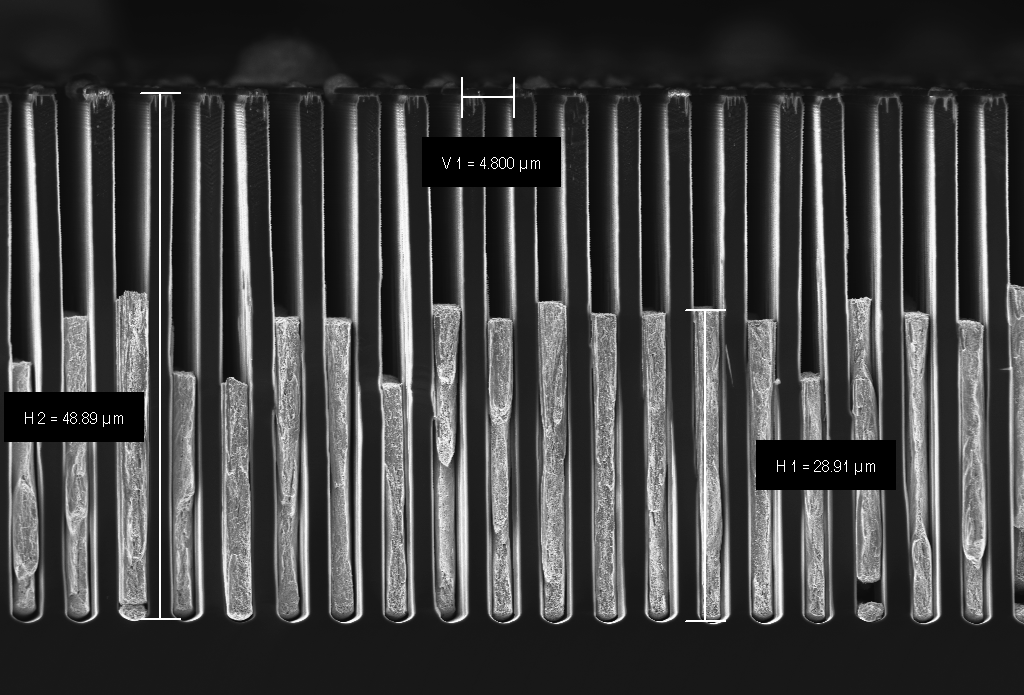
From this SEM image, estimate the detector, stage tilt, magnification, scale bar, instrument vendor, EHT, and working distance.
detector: InLens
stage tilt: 0°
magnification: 3.96 K X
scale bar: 10000 nm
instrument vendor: Zeiss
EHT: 5 kV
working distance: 4.7 mm